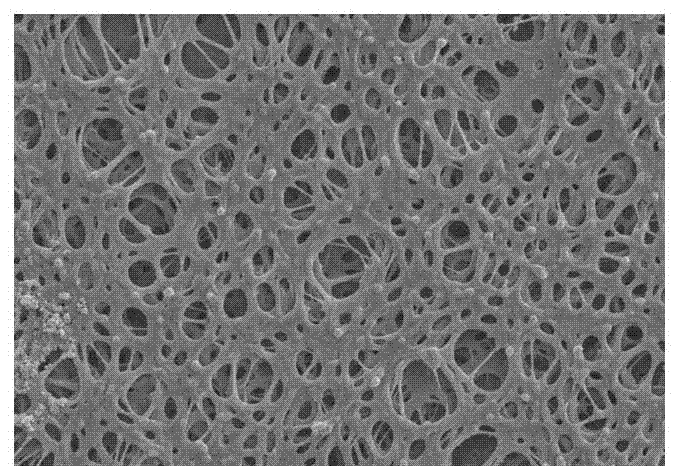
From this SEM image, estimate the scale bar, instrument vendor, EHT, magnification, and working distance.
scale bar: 10000 nm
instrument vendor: Hitachi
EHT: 5 kV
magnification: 5 K X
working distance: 11.2 mm